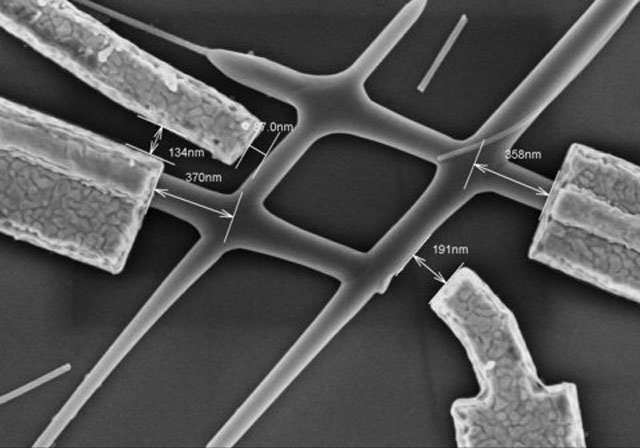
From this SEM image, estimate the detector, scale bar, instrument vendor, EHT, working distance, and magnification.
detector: SE(U,LA0)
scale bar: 1000 nm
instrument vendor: Hitachi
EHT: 3 kV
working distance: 3.9 mm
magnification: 45 K X